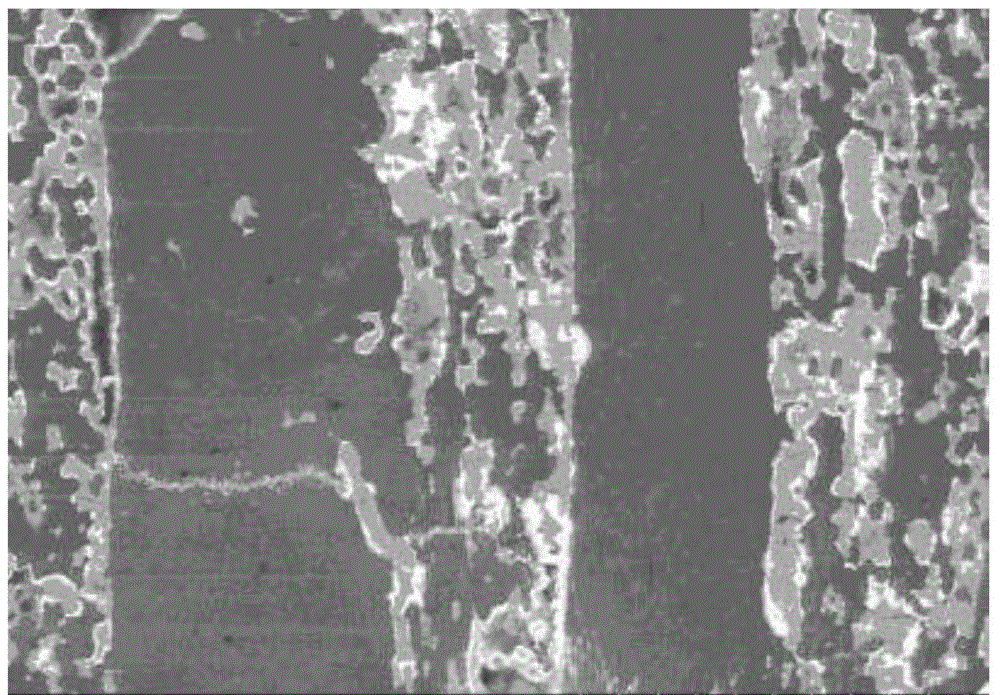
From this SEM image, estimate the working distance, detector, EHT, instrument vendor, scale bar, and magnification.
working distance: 10.4 mm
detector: YAGBSE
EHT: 15 kV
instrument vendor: Hitachi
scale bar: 500000 nm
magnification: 0.1 K X